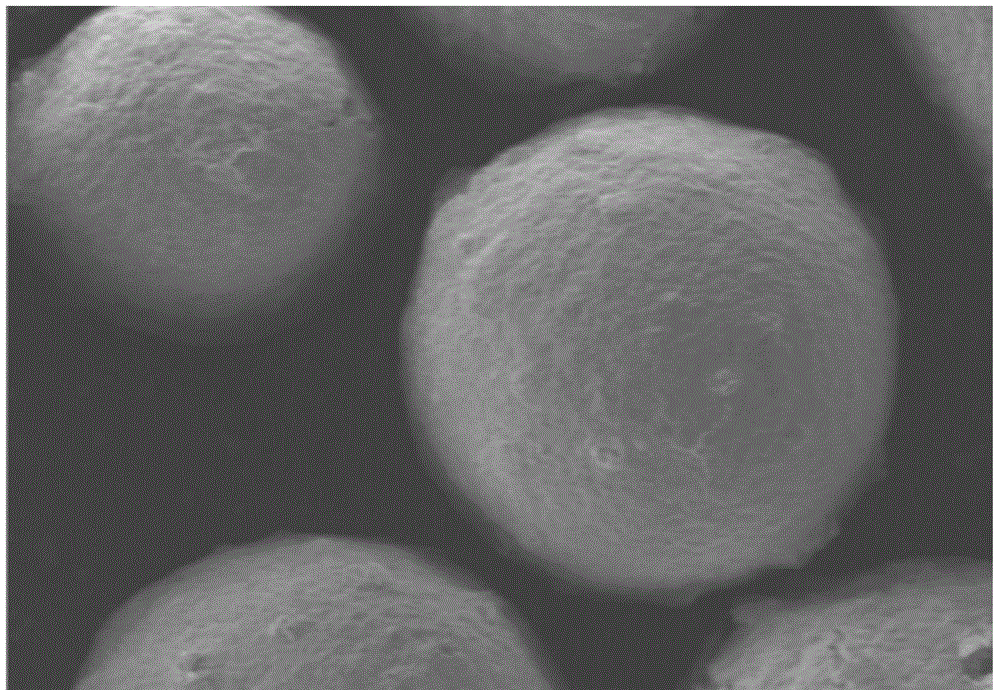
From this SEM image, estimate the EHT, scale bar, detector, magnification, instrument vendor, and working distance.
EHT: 5 kV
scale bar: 400000 nm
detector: SE(M)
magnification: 0.12 K X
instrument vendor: Hitachi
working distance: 16.2 mm